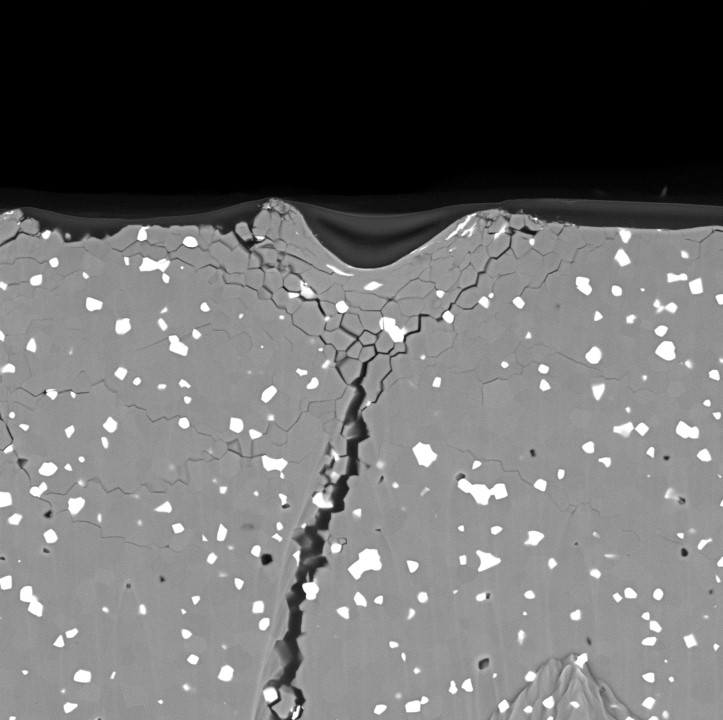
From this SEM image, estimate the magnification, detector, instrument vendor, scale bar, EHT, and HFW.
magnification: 2 K X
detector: BSD Full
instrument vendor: other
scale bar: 30000 nm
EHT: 15 kV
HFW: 134 µm